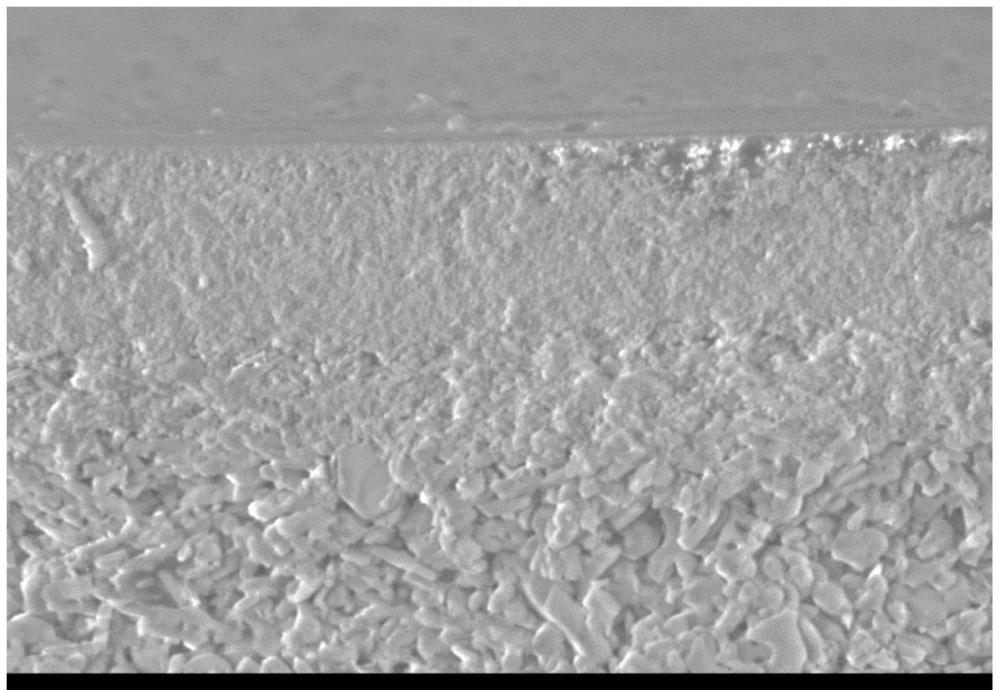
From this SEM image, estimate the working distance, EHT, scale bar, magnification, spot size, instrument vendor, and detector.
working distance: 14.2 mm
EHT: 15 kV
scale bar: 10000 nm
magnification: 2 K X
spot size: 12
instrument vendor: JEOL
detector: SE